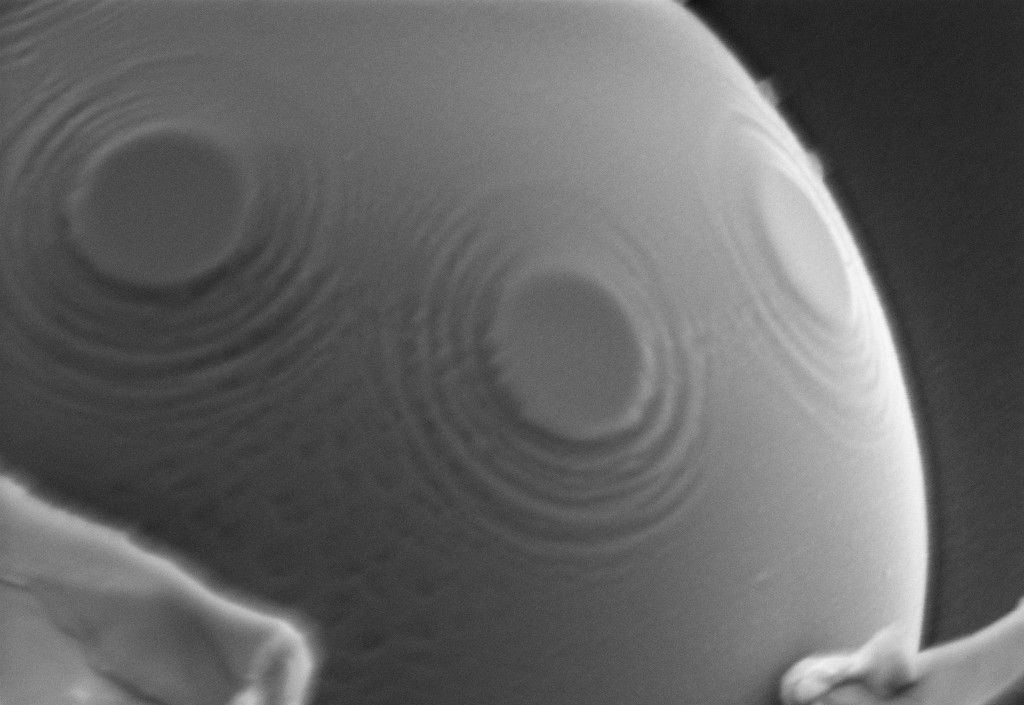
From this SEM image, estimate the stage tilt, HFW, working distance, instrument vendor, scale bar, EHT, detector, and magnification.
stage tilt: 0°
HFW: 3.376 µm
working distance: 4.5 mm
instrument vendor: Zeiss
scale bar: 200 nm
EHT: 5 kV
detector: InLens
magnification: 33.87 K X